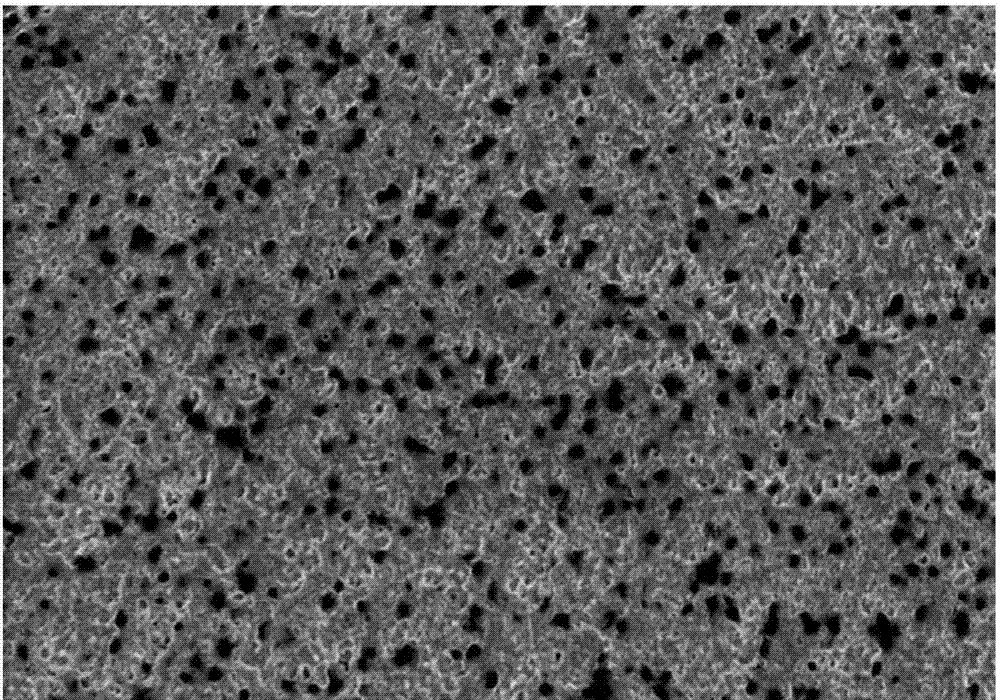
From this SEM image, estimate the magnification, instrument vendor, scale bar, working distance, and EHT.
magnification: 10 K X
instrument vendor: Hitachi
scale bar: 5000 nm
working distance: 7.2 mm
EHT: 1 kV